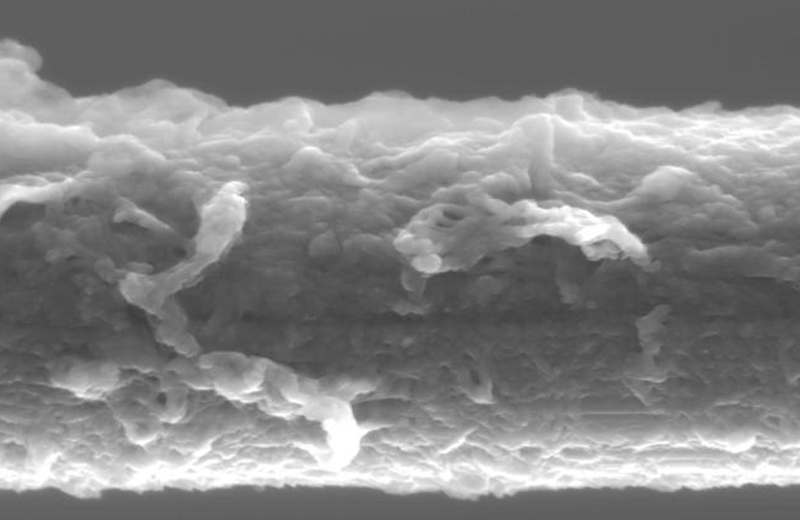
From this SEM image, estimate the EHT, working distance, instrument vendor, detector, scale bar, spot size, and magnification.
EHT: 8 kV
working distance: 6.1 mm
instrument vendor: FEI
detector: SE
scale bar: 2000 nm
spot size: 3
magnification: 10 K X